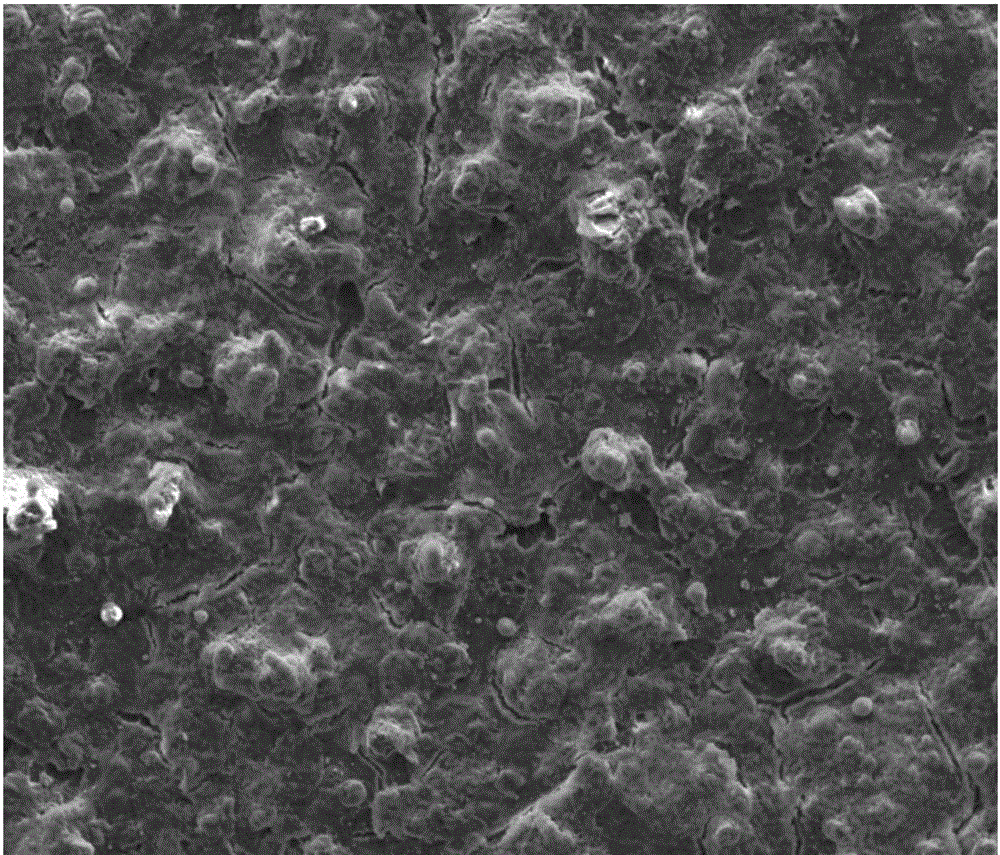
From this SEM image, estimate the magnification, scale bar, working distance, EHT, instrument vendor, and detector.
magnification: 1 K X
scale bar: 100000 nm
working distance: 4.7 mm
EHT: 10 kV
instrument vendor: FEI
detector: ETD